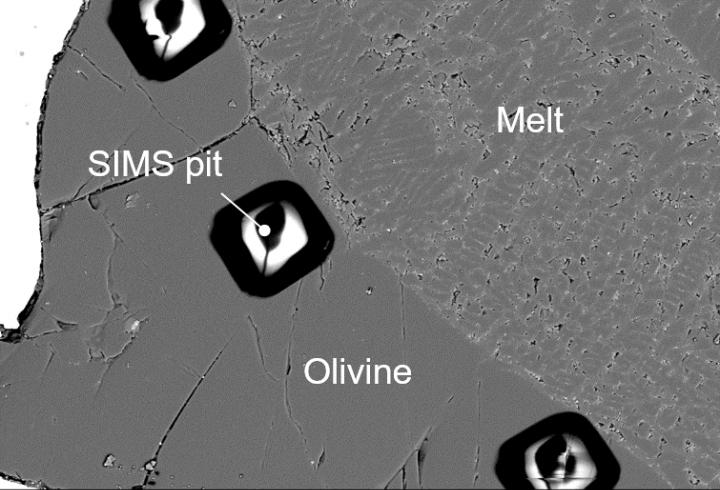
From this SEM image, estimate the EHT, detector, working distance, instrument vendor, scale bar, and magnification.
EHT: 15 kV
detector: BEC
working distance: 10 mm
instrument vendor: JEOL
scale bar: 50000 nm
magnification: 0.4 K X